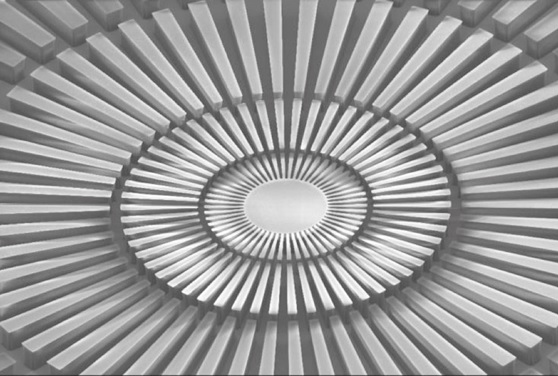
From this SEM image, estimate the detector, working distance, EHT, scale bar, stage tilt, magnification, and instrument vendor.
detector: SE2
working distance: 20.7 mm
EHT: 10 kV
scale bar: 10000 nm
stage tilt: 50°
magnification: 0.5 K X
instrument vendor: Zeiss